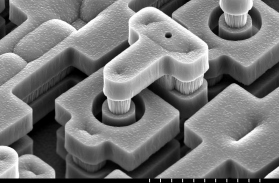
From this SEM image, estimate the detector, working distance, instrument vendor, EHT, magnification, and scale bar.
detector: SE(M)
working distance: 12.5 mm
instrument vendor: Hitachi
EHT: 10 kV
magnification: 5 K X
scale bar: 10000 nm